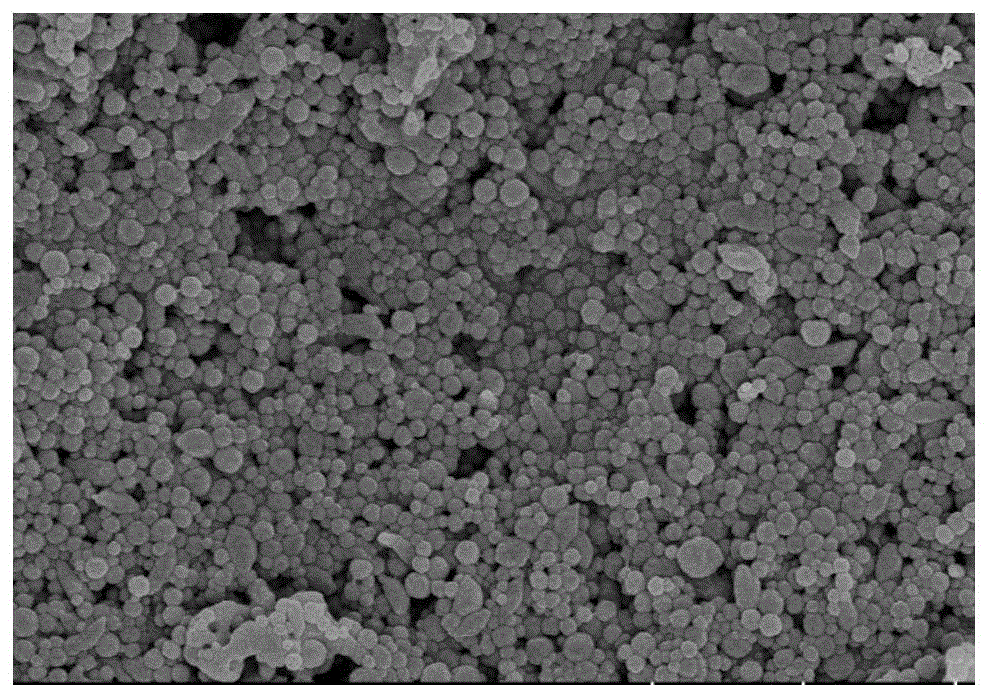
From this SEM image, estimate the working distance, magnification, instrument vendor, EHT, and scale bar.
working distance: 3.4 mm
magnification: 20 K X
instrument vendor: Hitachi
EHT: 5 kV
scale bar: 2000 nm